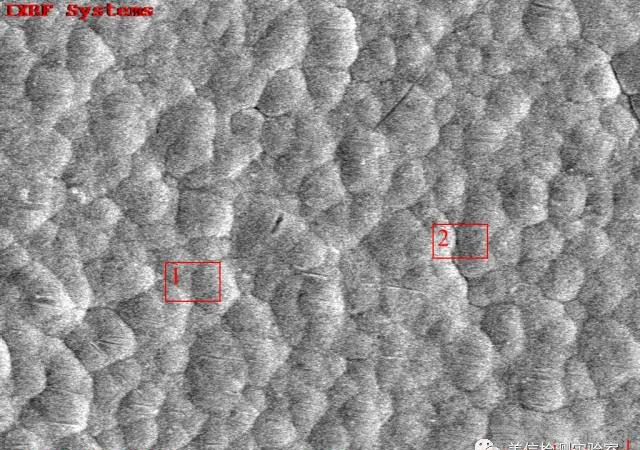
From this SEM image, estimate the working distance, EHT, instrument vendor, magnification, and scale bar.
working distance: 10.1 mm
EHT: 15 kV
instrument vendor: other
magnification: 3 K X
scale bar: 10000 nm